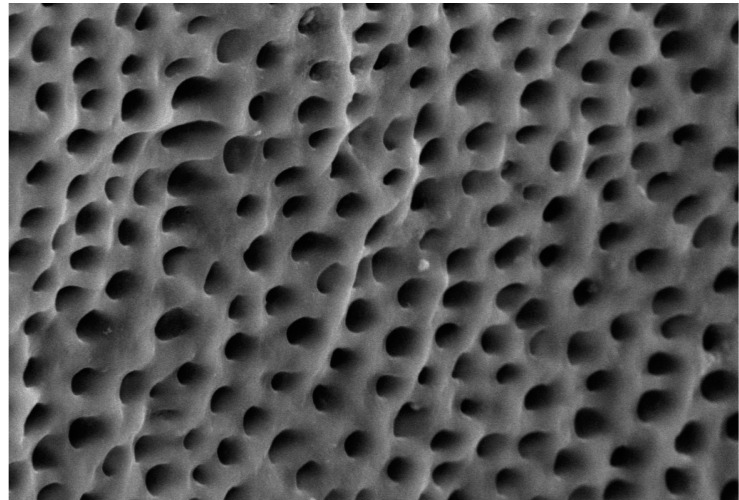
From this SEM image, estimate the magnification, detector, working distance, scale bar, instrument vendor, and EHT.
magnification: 5 K X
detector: SE1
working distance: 8.5 mm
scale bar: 3000 nm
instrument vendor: Zeiss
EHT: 15 kV